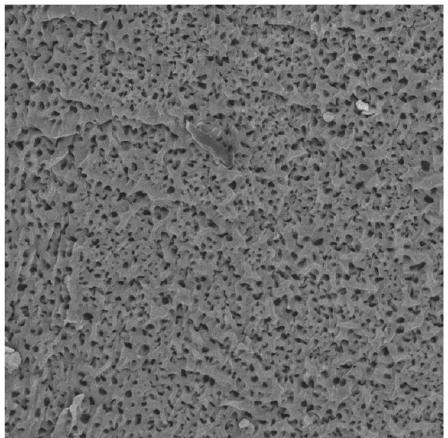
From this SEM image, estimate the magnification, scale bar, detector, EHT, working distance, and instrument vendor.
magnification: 1 K X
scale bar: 50000 nm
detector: BSE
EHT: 20 kV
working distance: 17.58 mm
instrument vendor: Tescan